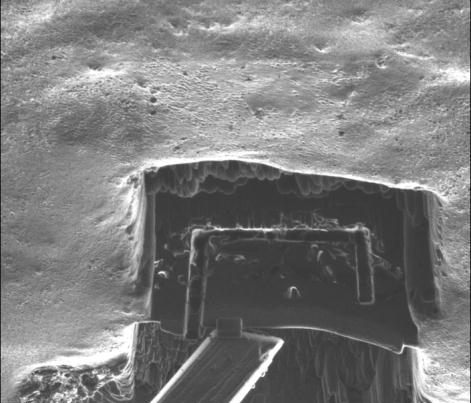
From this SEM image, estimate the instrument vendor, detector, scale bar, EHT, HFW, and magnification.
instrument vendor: FEI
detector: ETD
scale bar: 10000 nm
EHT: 30 kV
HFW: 39.4 µm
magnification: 6.5 K X